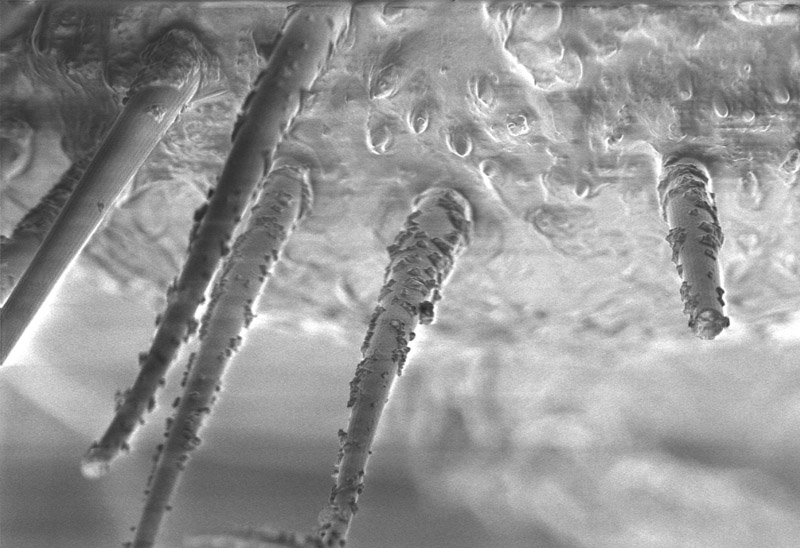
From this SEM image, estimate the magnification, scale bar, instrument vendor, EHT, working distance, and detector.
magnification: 1 K X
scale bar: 20000 nm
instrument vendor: JEOL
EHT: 2 kV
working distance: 8 mm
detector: ECD-1